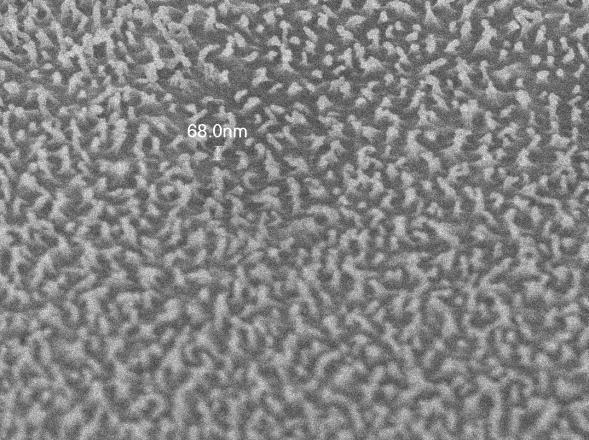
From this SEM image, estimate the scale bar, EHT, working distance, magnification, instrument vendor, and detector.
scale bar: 100 nm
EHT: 2 kV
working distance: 3.4 mm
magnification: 40 K X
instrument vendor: JEOL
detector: SEI